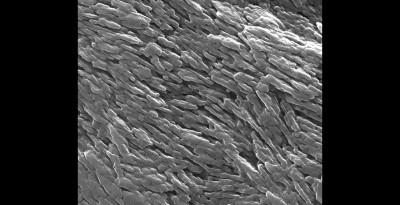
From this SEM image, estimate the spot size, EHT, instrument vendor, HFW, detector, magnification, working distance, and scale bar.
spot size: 3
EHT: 30 kV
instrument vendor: FEI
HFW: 2.98 µm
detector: ETD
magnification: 100 K X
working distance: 10.1 mm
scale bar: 1000 nm